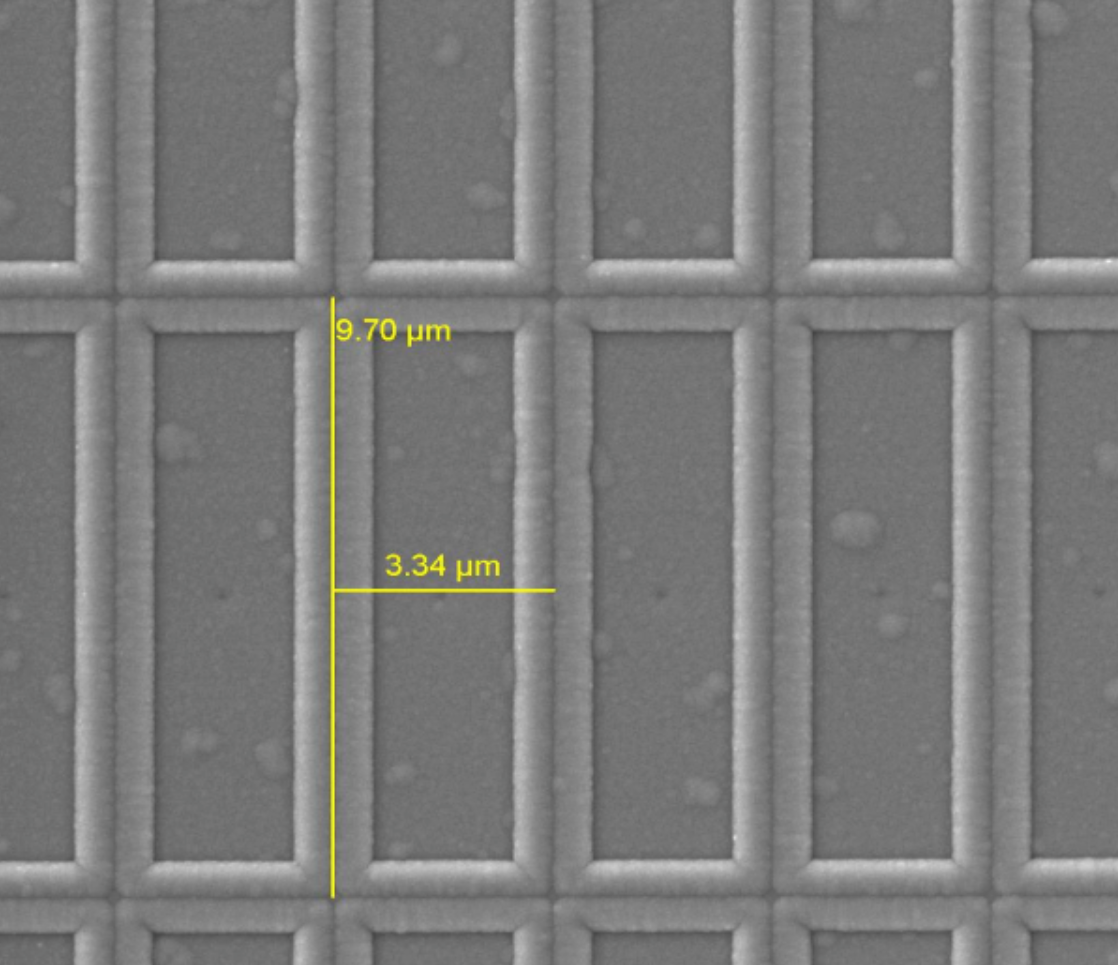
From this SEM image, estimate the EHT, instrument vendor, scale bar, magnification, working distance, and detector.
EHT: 5 kV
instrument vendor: other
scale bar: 3000 nm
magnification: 10 K X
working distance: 9.277 mm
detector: SE